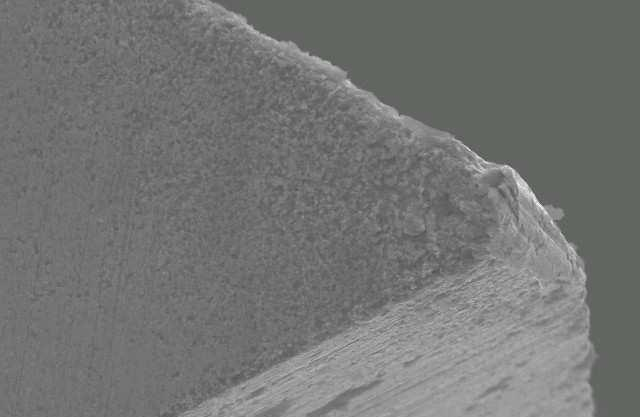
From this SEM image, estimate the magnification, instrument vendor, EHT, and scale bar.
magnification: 1 K X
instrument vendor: JEOL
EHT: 20 kV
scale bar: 10000 nm